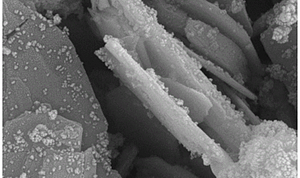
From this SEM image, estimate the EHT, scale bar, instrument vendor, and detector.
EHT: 10 kV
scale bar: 300 nm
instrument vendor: Zeiss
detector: SE2